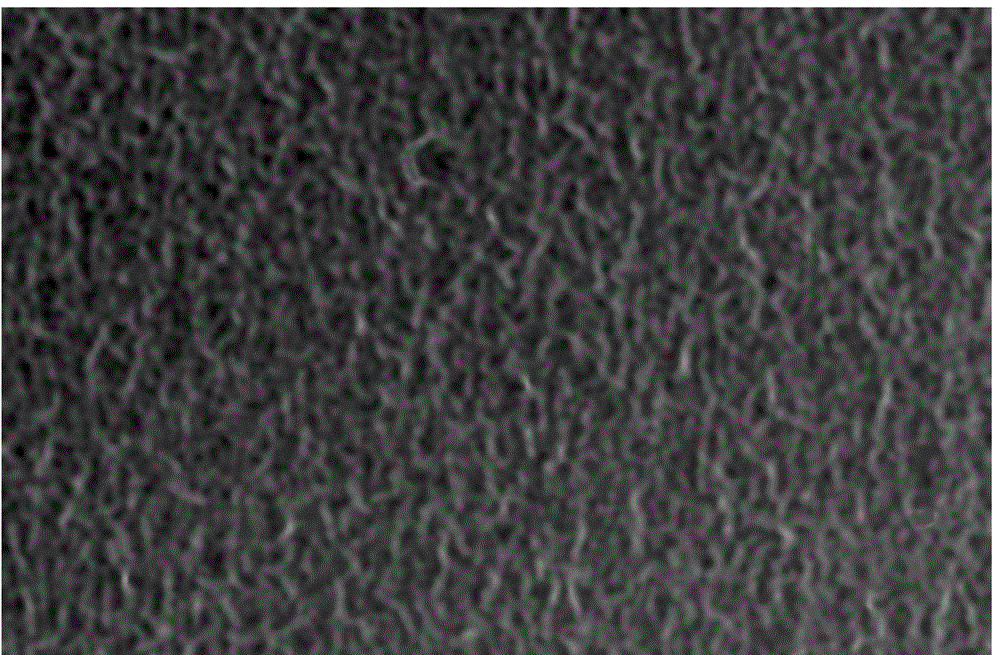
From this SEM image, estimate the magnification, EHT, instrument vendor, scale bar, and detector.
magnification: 10 K X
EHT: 20 kV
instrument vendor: JEOL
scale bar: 1000 nm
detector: SEI